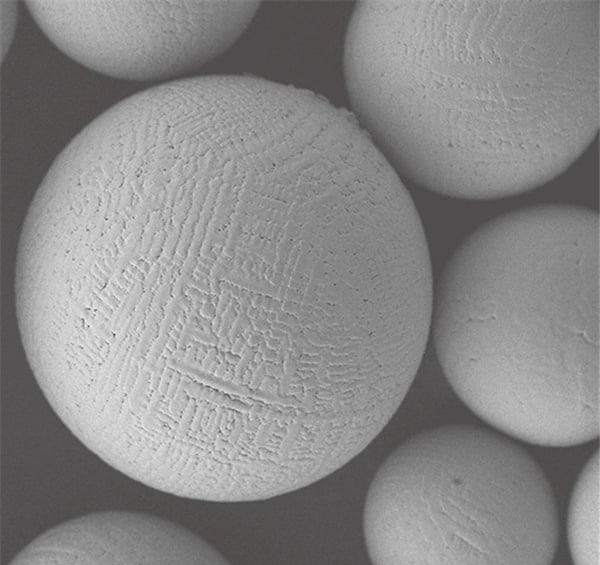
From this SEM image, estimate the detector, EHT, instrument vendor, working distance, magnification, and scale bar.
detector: LEI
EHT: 5 kV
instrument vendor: JEOL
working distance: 9 mm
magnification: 1 K X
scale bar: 10000 nm